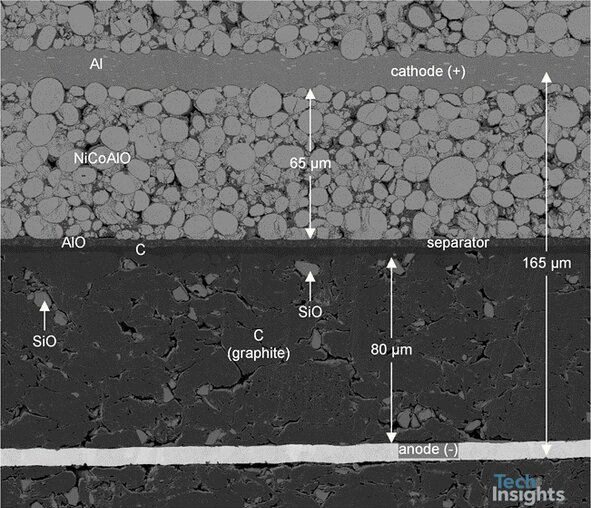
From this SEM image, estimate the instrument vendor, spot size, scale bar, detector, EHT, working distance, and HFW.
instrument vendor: FEI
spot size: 3.5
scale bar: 100000 nm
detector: CBS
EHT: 5 kV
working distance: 12.9 mm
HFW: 254 µm